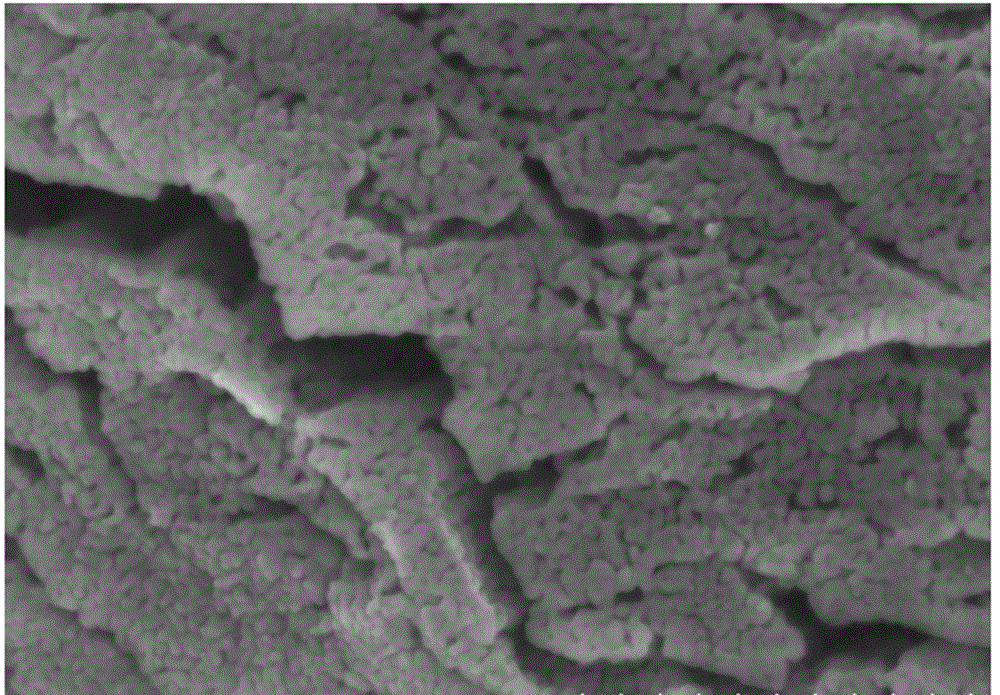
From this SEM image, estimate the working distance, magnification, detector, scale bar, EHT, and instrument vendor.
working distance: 5.7 mm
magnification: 100 K X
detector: SE(M)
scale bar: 500 nm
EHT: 3 kV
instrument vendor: Hitachi